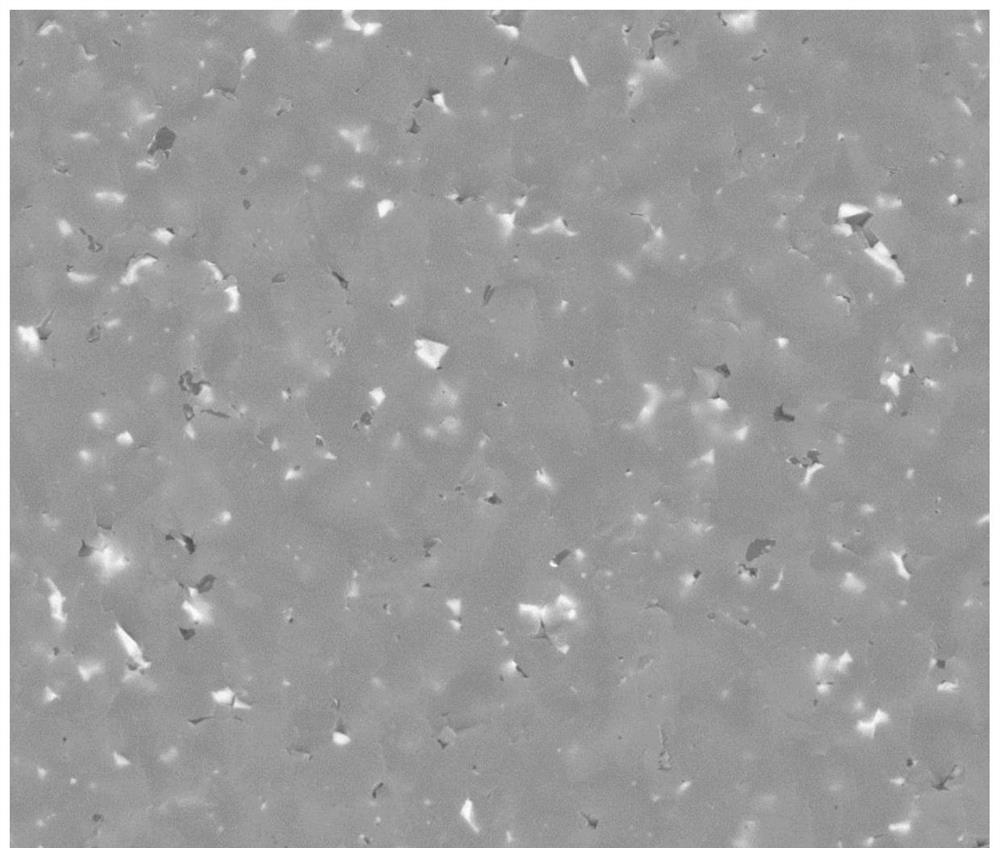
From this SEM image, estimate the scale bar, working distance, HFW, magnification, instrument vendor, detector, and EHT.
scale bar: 20000 nm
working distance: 9 mm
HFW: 59.7 µm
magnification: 5 K X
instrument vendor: FEI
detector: BSED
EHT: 20 kV